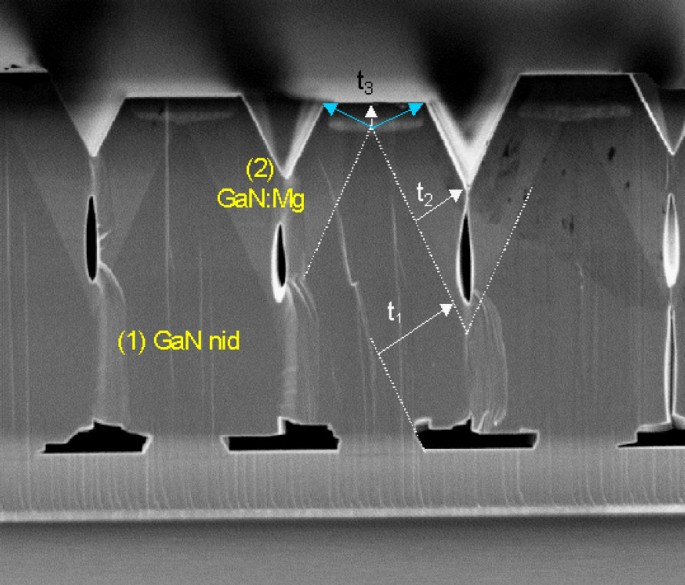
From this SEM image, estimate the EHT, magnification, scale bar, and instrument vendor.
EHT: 5 kV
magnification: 3.3 K X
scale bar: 10000 nm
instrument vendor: JEOL